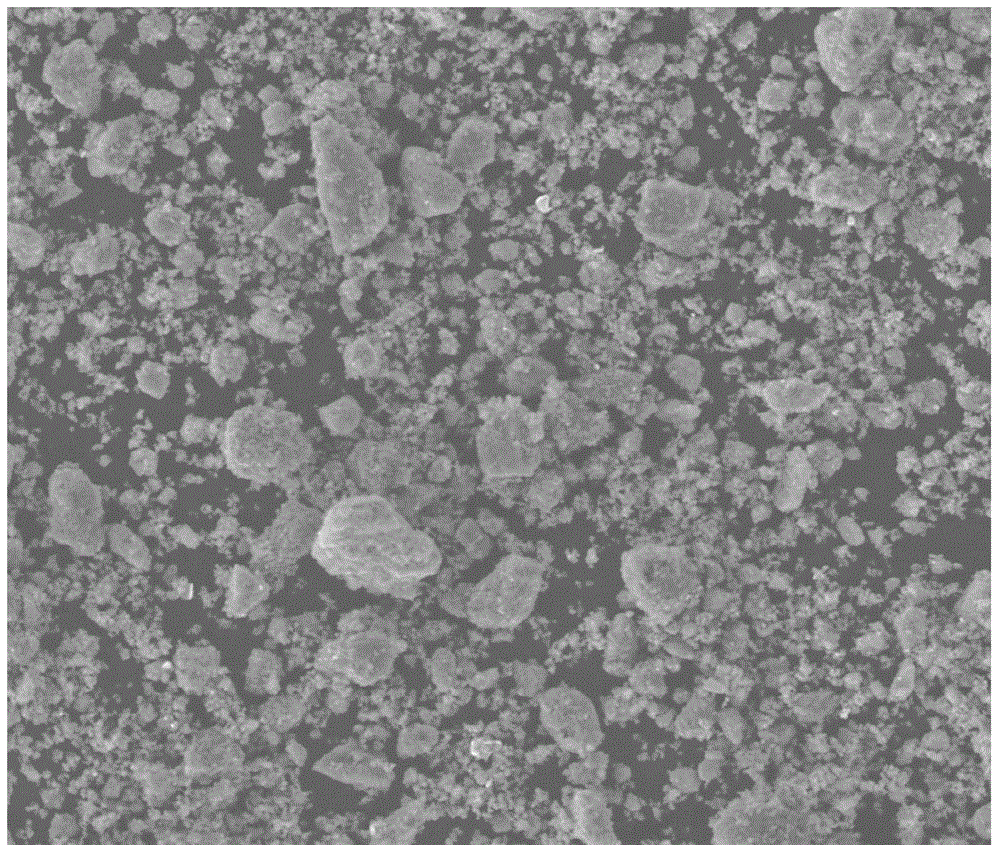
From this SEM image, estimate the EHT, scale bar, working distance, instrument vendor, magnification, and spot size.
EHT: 20 kV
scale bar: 100000 nm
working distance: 9.9 mm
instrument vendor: FEI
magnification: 0.5 K X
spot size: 4.5